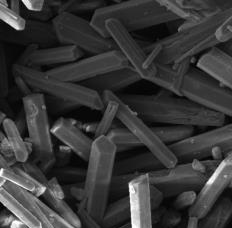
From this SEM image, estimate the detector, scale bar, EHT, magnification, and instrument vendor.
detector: SE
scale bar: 20000 nm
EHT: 15 kV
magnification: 1 K X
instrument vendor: Tescan